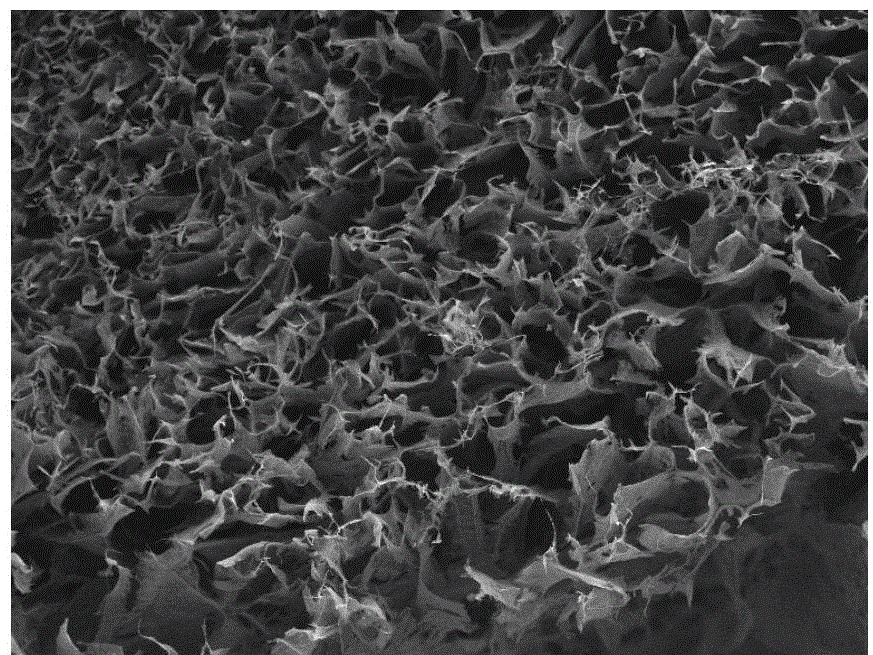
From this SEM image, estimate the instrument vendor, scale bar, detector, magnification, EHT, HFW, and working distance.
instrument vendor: Tescan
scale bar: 500000 nm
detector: SE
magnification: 0.1 K X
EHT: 20 kV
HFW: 2080 µm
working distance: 17.75 mm